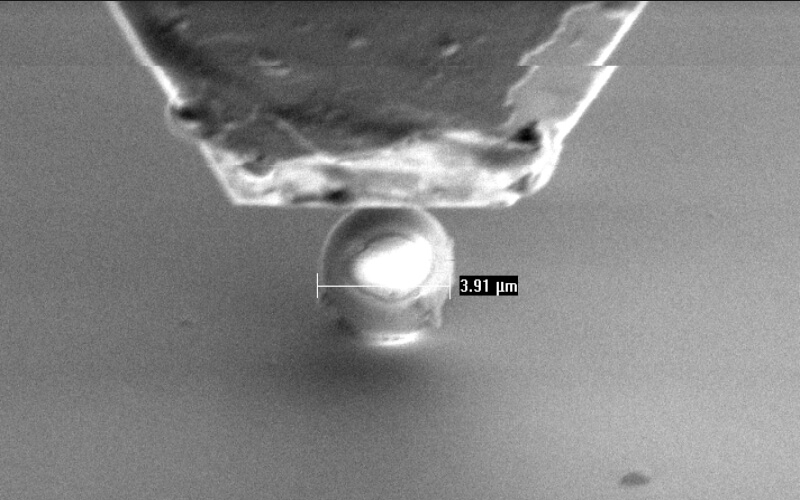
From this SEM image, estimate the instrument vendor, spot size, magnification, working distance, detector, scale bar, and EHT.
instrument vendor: FEI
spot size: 3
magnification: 5 K X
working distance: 10 mm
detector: SE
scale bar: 5000 nm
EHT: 3 kV